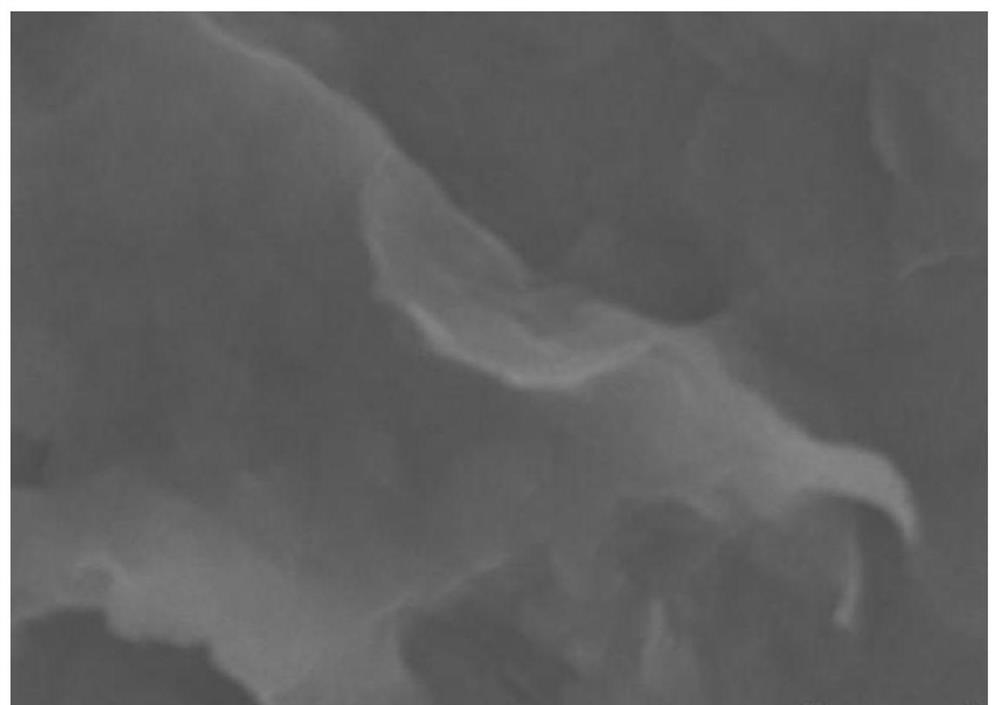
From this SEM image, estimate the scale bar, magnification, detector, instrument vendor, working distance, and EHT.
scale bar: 500 nm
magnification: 80 K X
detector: SE(UL)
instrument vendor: Hitachi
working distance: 9.6 mm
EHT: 5 kV